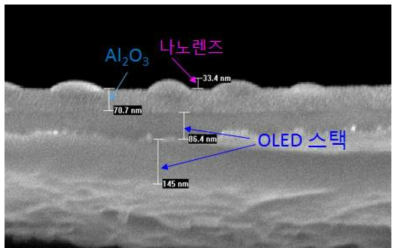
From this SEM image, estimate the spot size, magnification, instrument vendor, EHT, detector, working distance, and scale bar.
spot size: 3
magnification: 100 K X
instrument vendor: FEI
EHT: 10 kV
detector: TLD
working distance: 5.4 mm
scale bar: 200 nm